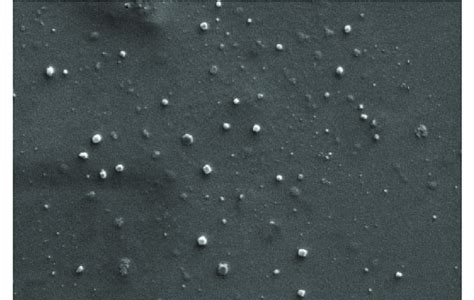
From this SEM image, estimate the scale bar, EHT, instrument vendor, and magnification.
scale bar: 1000 nm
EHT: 26 kV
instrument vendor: other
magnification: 40 K X